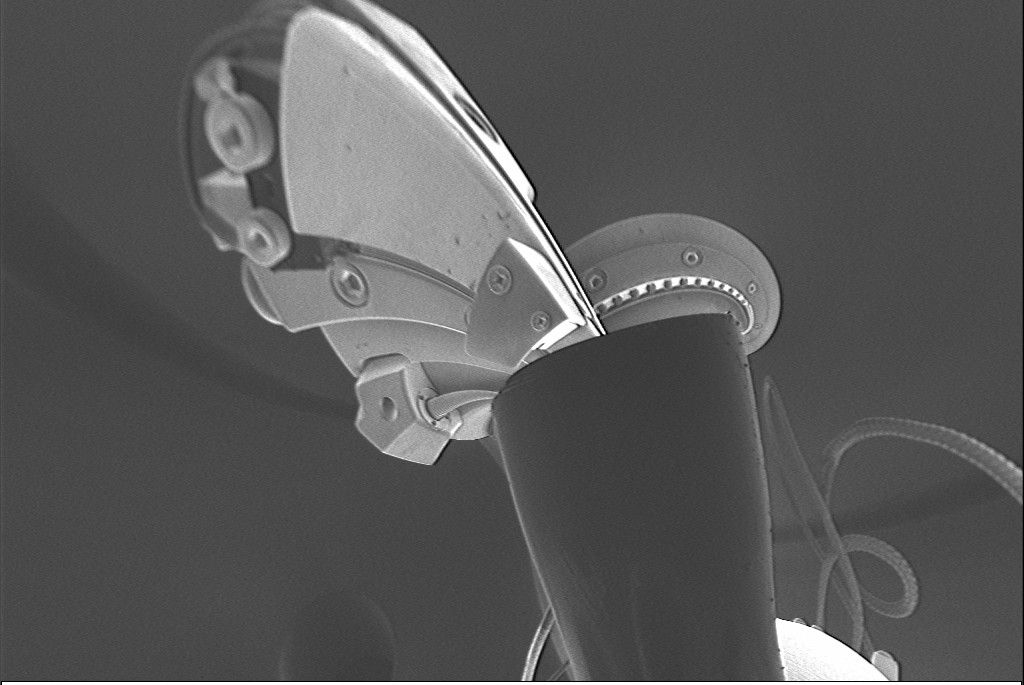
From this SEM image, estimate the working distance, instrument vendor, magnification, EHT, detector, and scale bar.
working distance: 4.8 mm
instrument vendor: Zeiss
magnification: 0.467 K X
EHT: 1 kV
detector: SE2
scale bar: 20000 nm